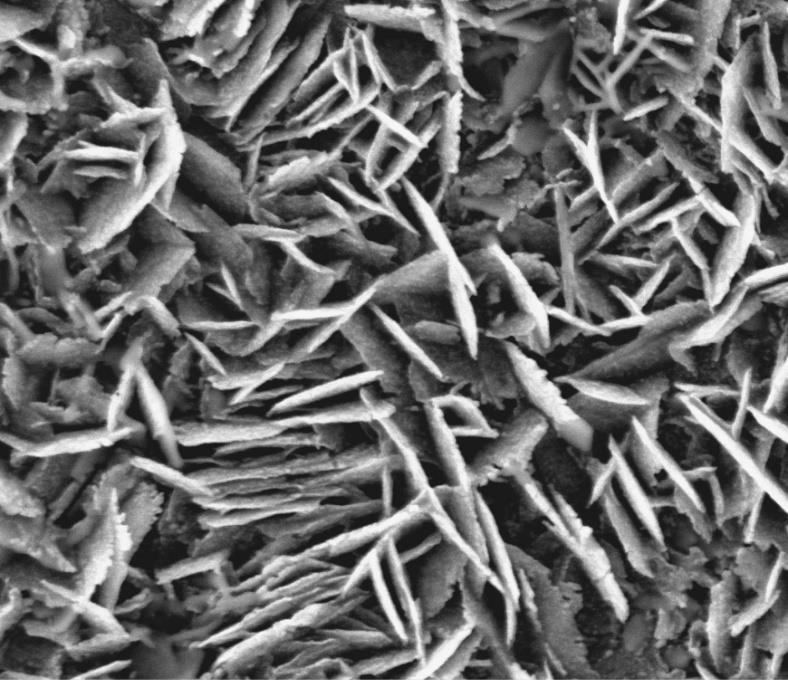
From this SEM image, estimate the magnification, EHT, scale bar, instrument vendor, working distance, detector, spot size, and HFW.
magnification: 10 K X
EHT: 10 kV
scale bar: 5000 nm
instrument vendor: FEI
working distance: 4.6 mm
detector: ETD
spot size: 3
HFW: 14.9 µm